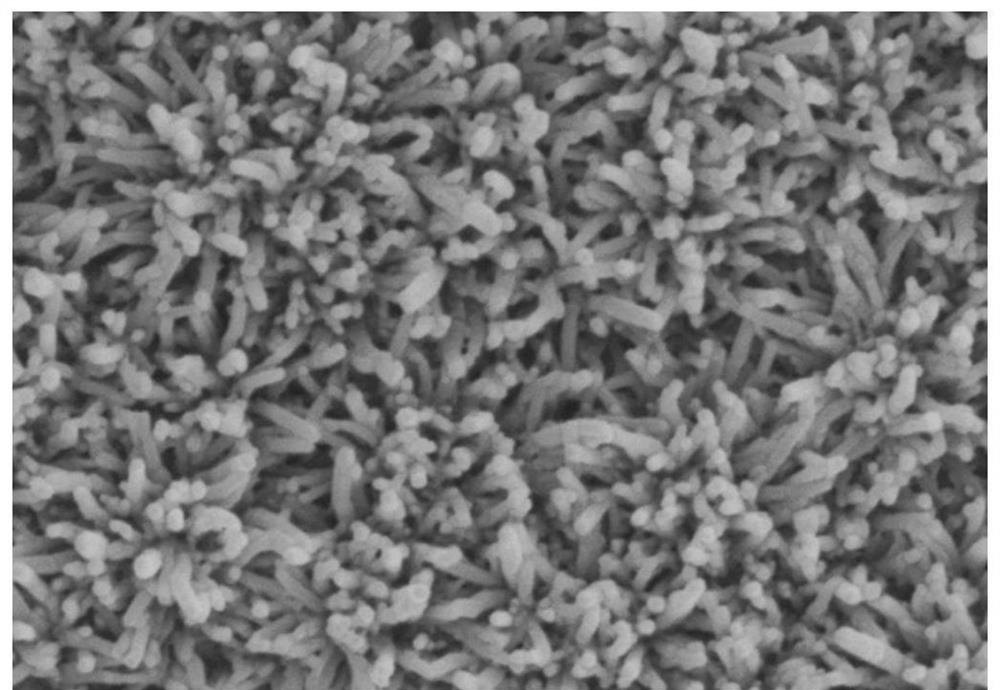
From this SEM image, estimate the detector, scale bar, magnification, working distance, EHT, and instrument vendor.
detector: SE(M)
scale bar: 1000 nm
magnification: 50 K X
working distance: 9.5 mm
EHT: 3 kV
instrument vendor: Hitachi